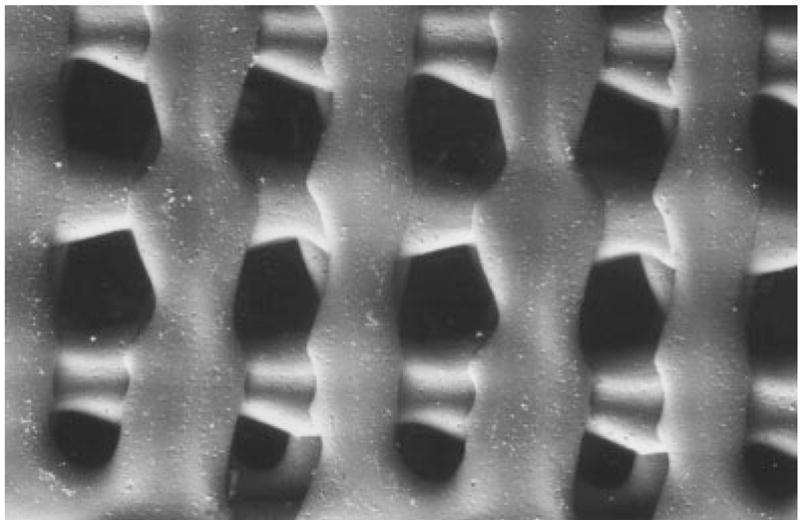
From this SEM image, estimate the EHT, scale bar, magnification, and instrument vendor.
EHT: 25 kV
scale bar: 2000000 nm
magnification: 0.025 K X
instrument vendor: JEOL